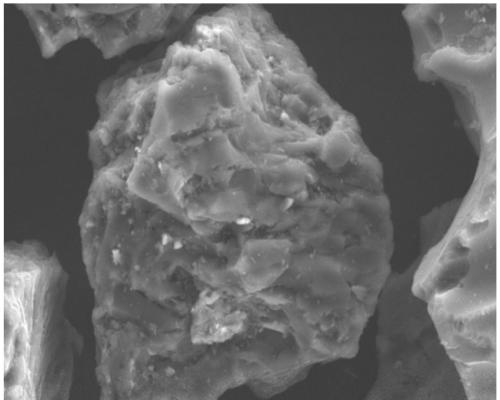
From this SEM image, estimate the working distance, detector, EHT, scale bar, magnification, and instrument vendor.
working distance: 7.7 mm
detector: SE2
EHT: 15 kV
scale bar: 10000 nm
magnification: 1 K X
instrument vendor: Zeiss